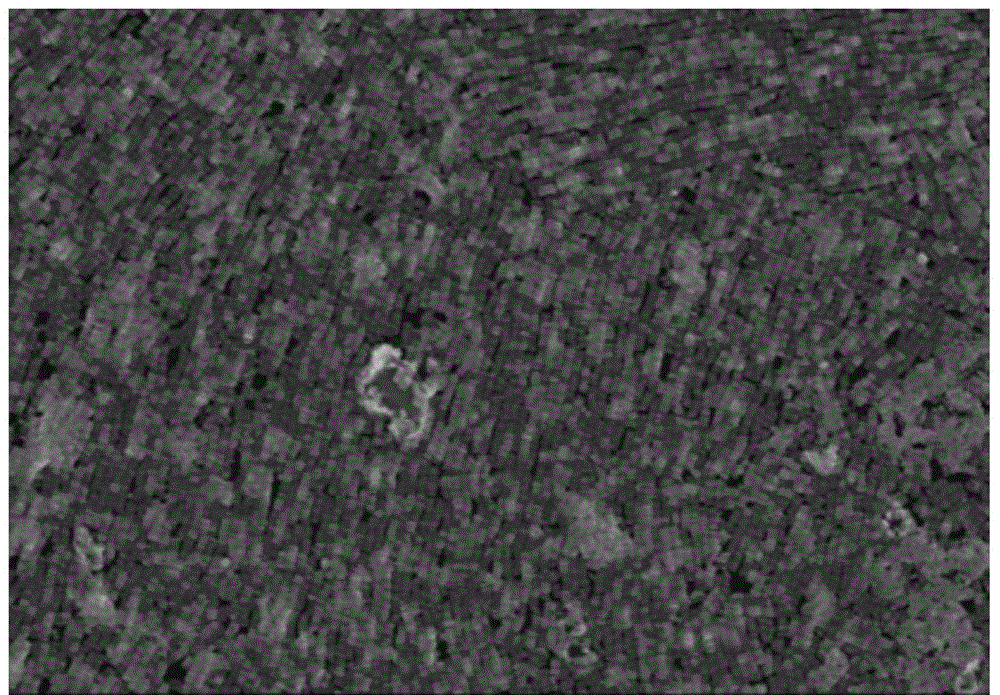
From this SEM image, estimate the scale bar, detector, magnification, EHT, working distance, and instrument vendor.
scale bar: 3000 nm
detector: SE(M)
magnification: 18 K X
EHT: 10 kV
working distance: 10 mm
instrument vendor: Hitachi